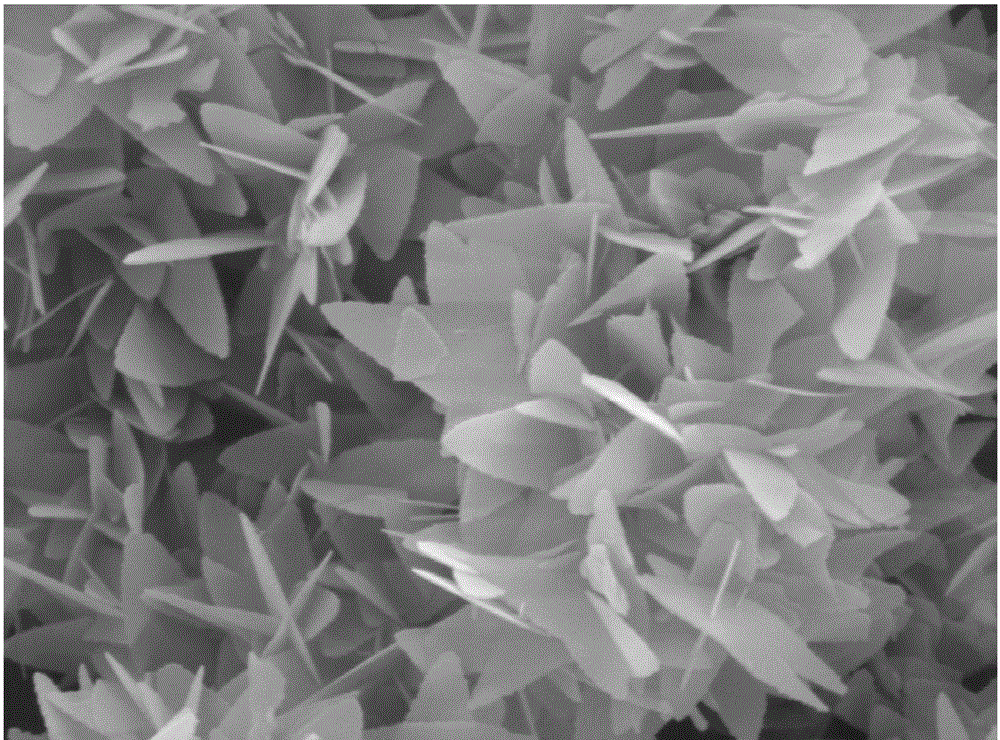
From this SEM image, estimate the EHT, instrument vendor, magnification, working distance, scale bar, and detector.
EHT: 8 kV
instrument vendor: JEOL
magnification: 10 K X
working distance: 10.1 mm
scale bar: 1000 nm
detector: LED